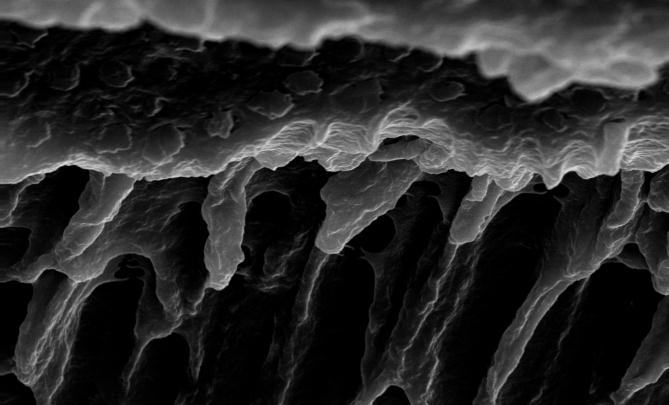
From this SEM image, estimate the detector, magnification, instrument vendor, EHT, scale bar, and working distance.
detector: SE1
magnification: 2.5 K X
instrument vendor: Zeiss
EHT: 20 kV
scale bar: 2000 nm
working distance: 12 mm